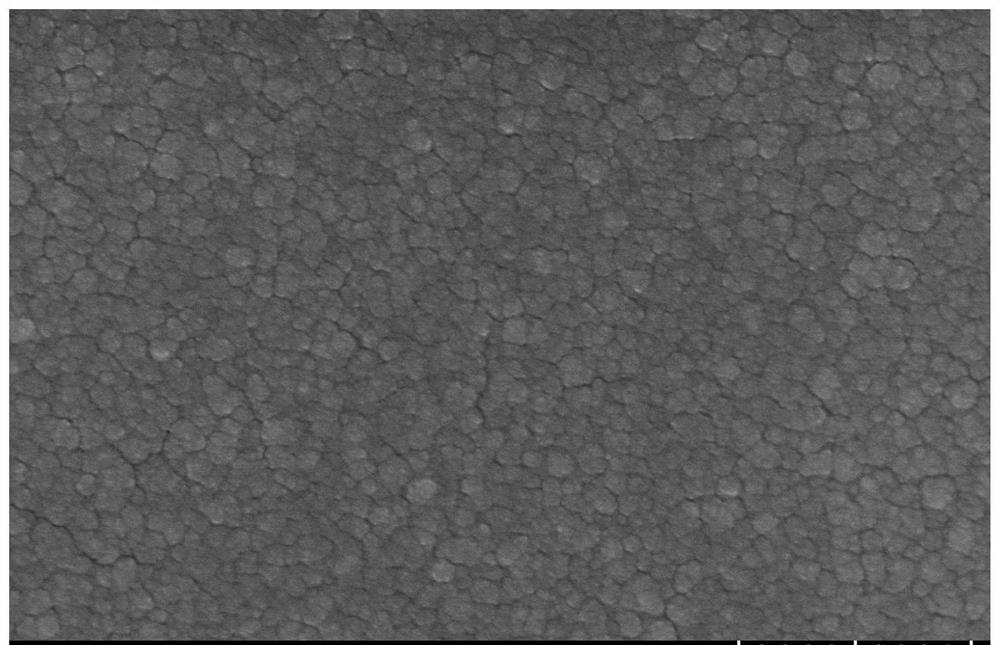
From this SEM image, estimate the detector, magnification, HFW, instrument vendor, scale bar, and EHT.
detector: SE(U)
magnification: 60.1 K X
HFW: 2.11 µm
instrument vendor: Hitachi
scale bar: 500 nm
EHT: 5 kV